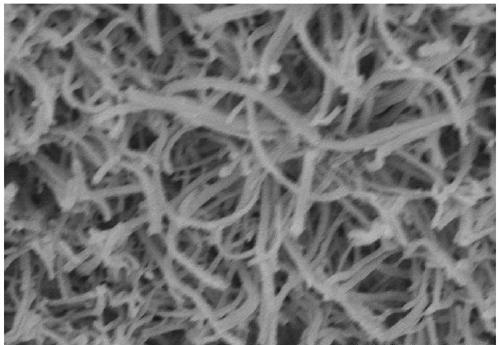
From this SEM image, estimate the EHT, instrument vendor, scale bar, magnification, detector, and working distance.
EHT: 10 kV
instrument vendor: Hitachi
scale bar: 4000 nm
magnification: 12 K X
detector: SE(U)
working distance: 8.8 mm